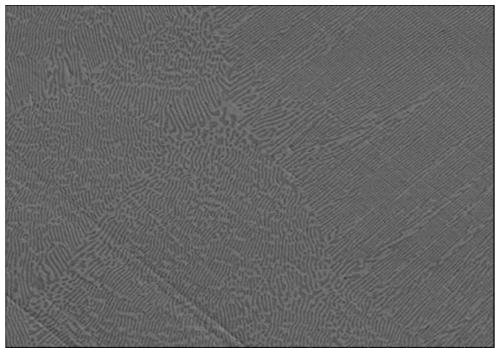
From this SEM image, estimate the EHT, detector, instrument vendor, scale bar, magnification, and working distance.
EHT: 15 kV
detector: SE(M,LA100)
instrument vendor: Hitachi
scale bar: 50000 nm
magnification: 1 K X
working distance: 9.1 mm